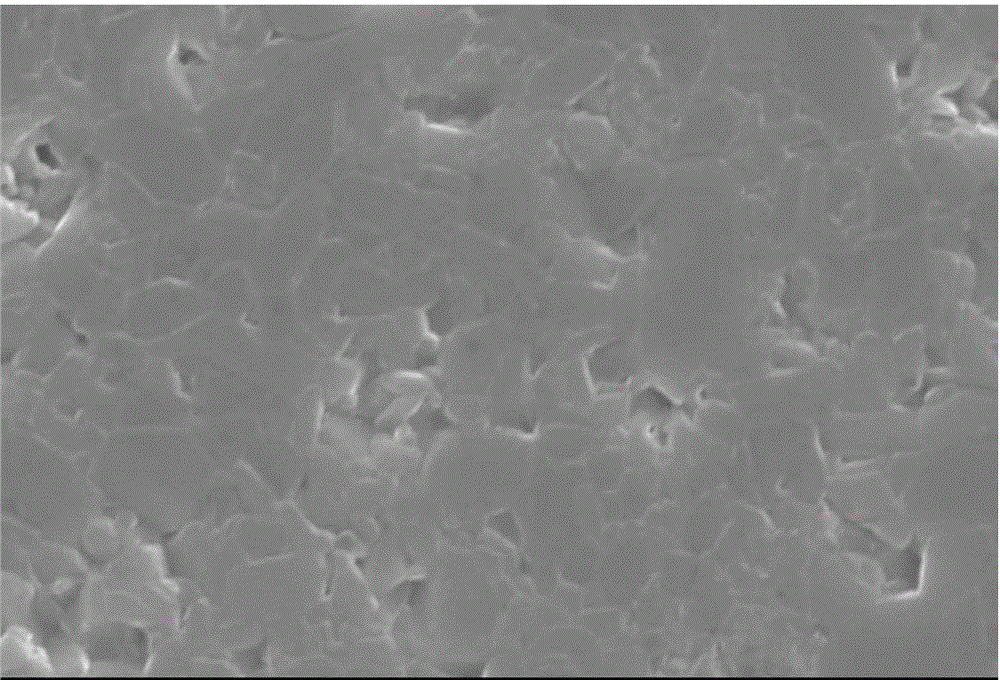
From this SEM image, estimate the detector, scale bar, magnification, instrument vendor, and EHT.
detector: SEI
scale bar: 10000 nm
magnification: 2.2 K X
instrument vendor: JEOL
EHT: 30 kV